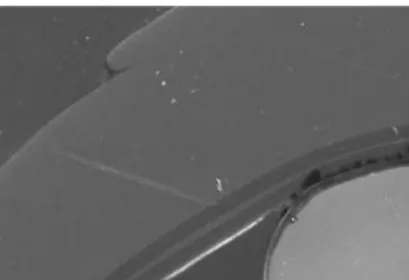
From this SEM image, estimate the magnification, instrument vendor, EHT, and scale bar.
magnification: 0.27 K X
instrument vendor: JEOL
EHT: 15 kV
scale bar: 50000 nm